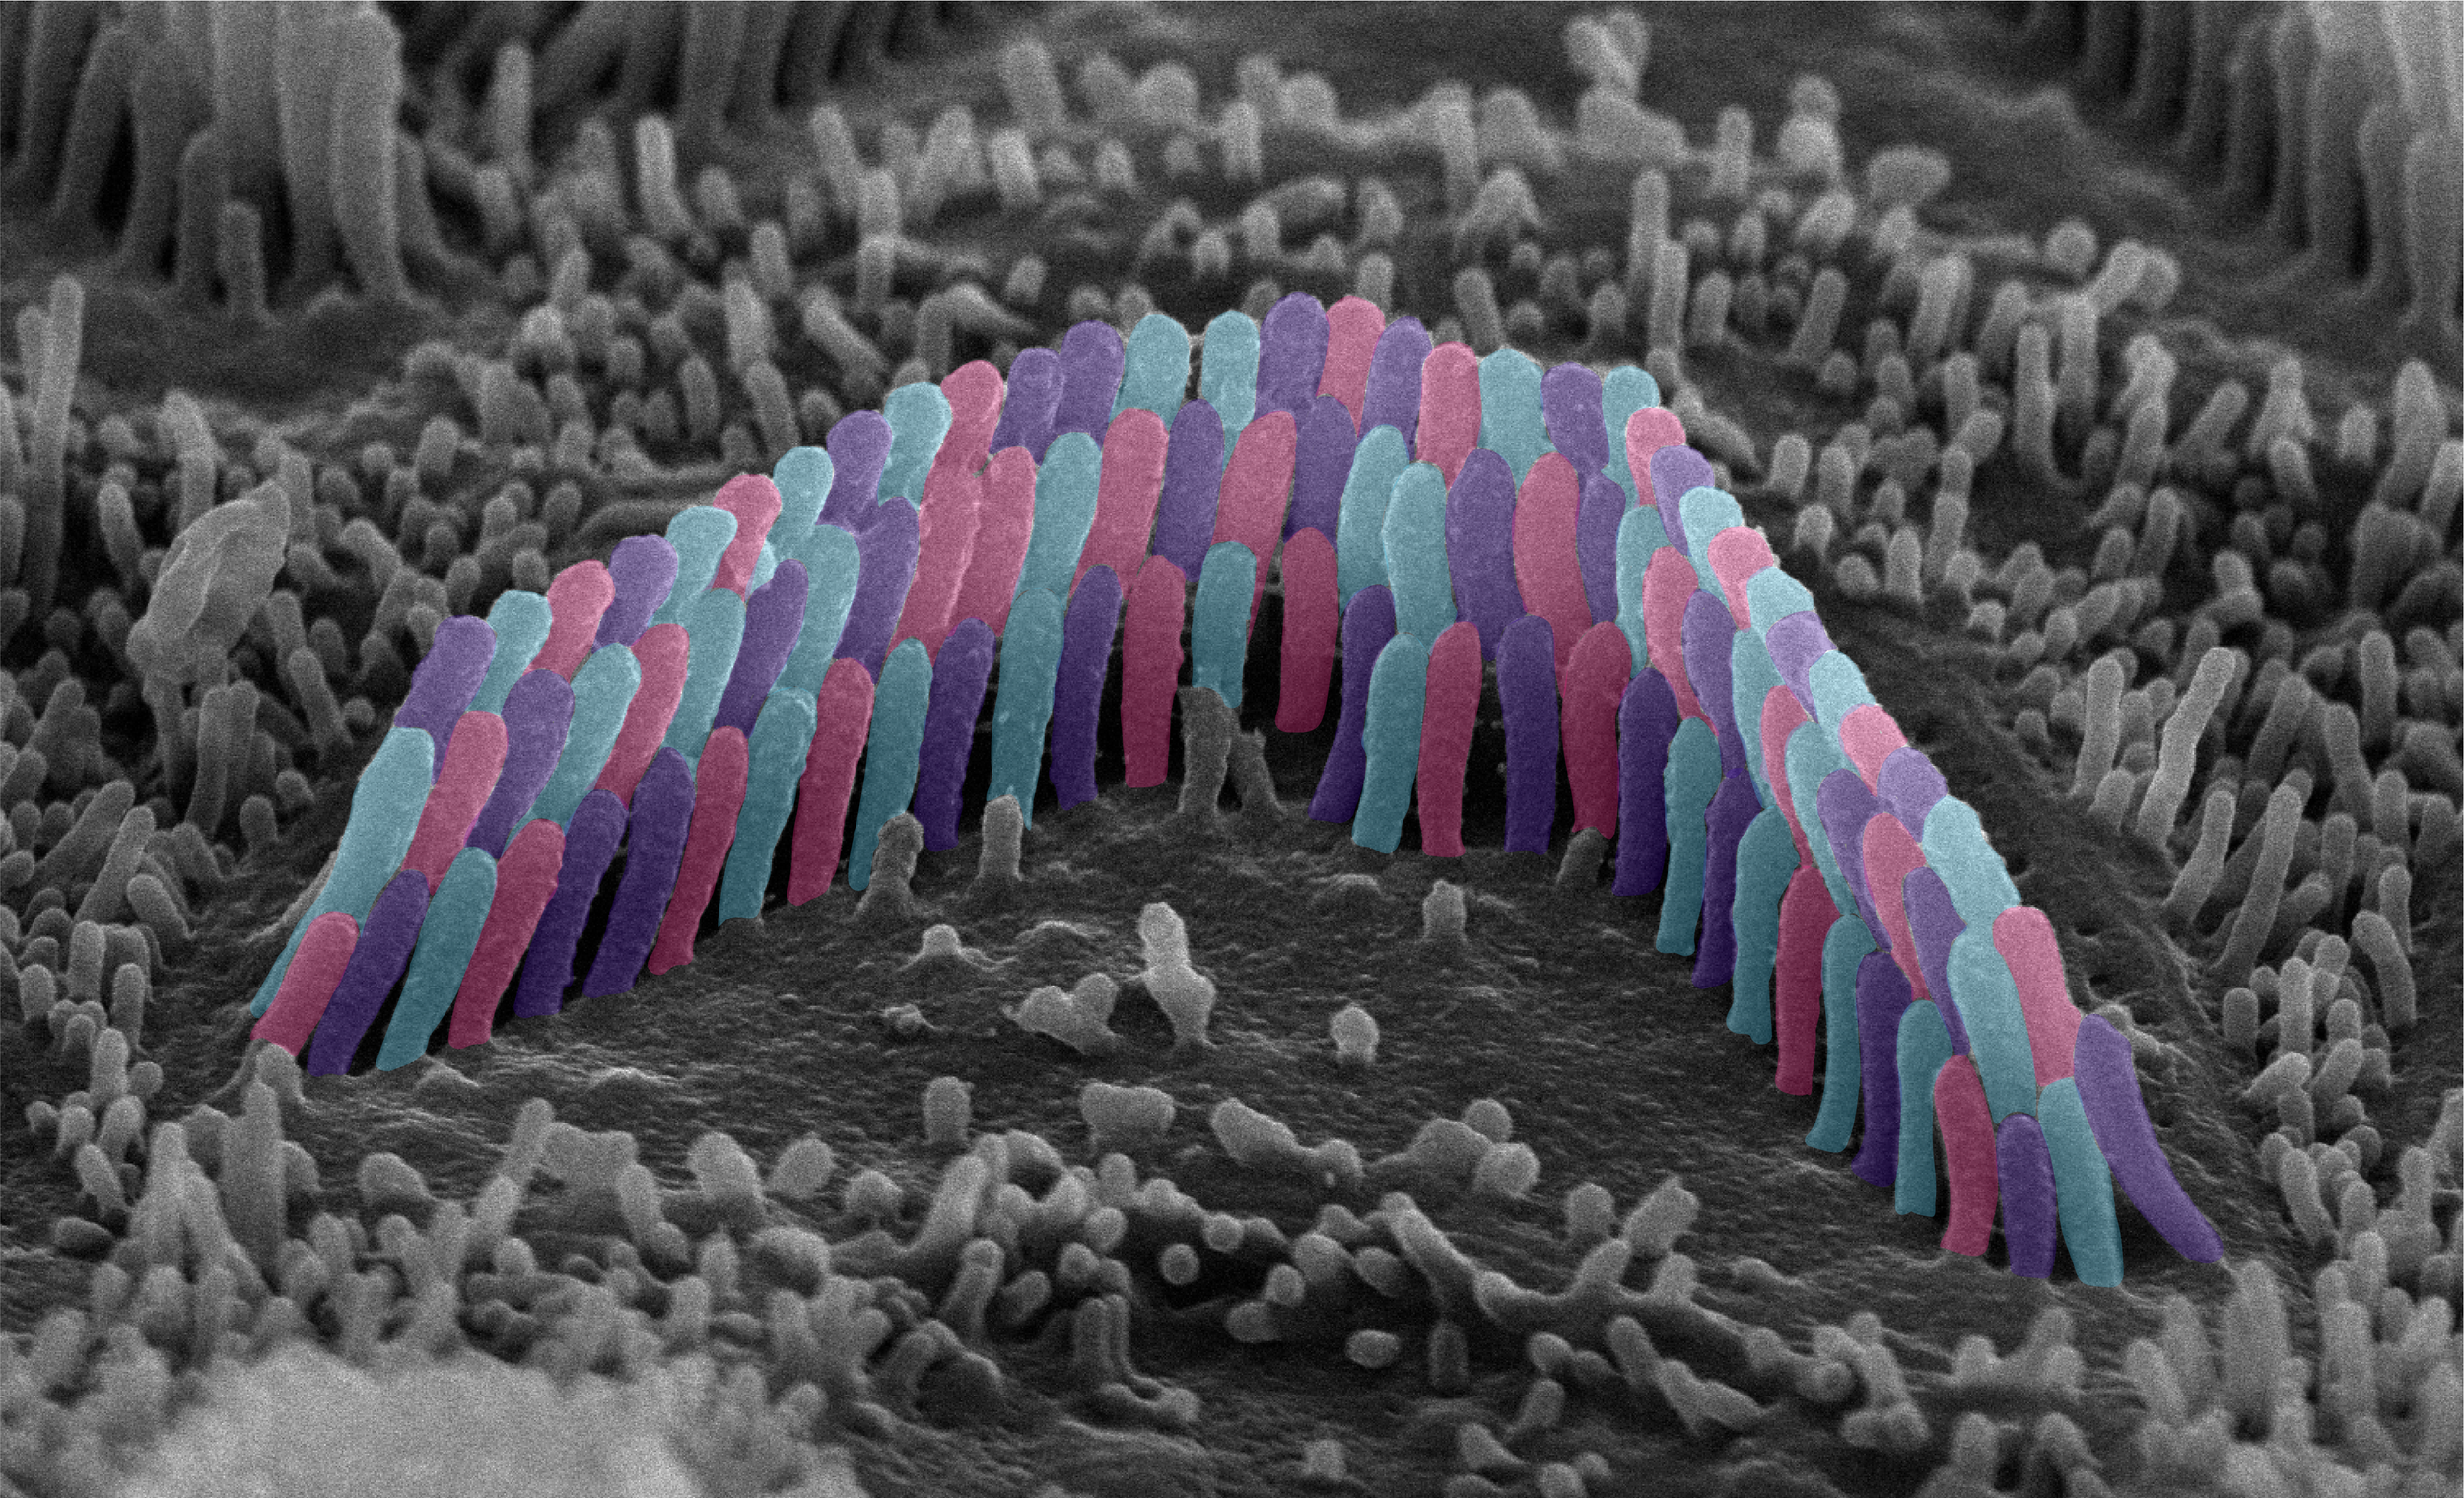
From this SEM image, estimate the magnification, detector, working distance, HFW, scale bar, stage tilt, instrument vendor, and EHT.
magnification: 25 K X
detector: TLD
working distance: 3.8 mm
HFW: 5.08 µm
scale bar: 1000 nm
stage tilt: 40°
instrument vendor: FEI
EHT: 10 kV